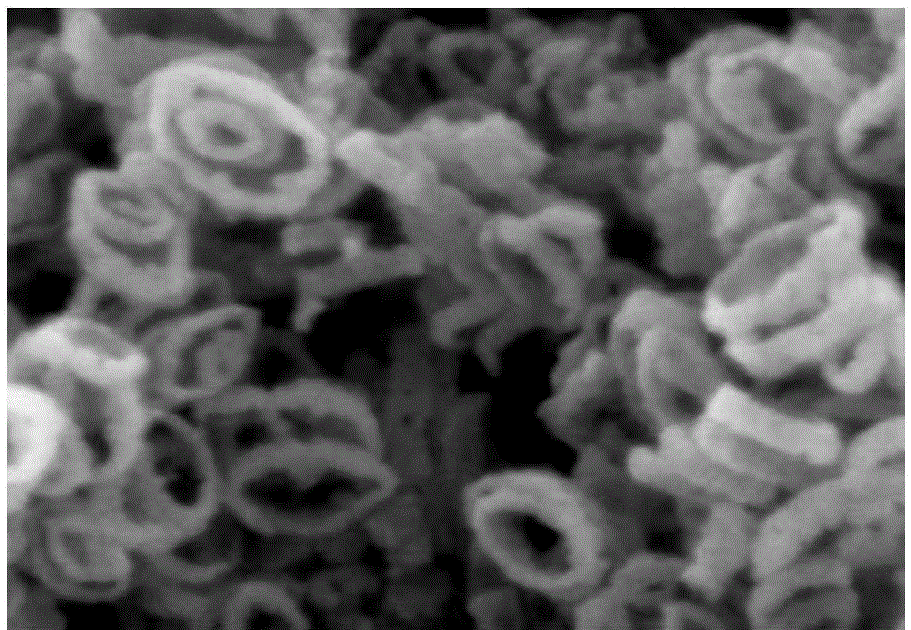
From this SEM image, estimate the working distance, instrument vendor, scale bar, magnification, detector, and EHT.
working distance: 6.9 mm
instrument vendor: Hitachi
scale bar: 300 nm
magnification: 150 K X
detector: SE(M)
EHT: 5 kV